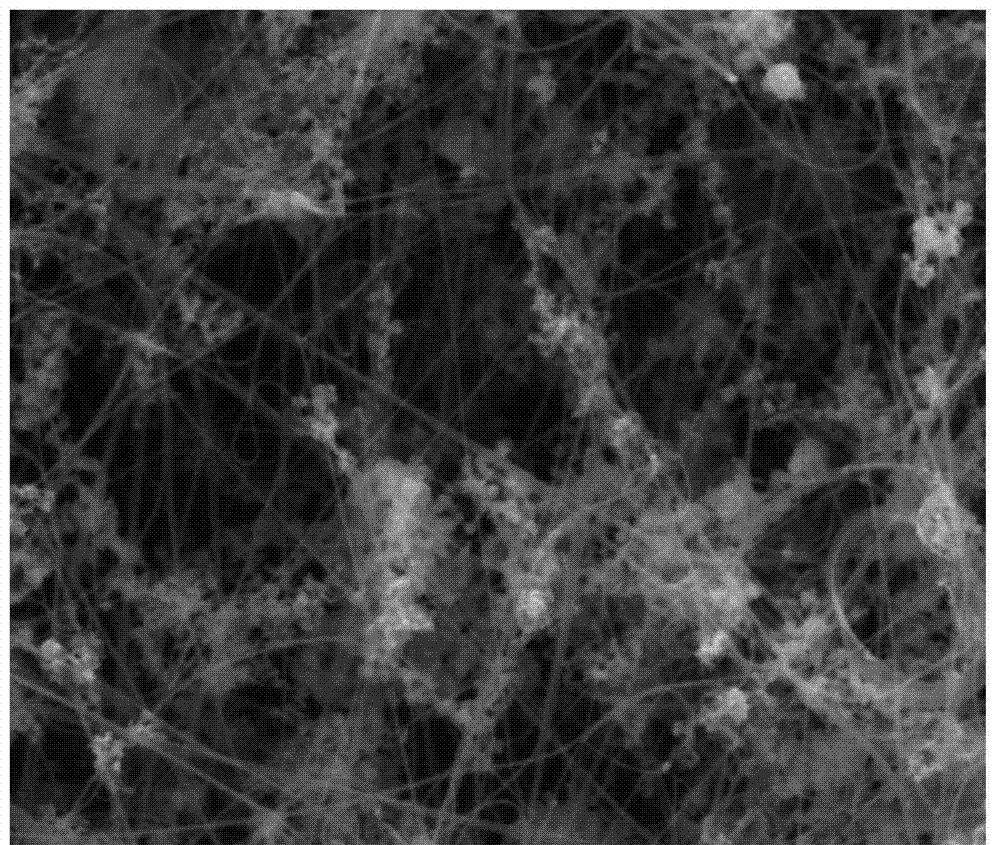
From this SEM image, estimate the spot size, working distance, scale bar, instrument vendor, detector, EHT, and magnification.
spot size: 3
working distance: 8.1 mm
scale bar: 1000 nm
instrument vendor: FEI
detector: ETD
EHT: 10 kV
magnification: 50 K X